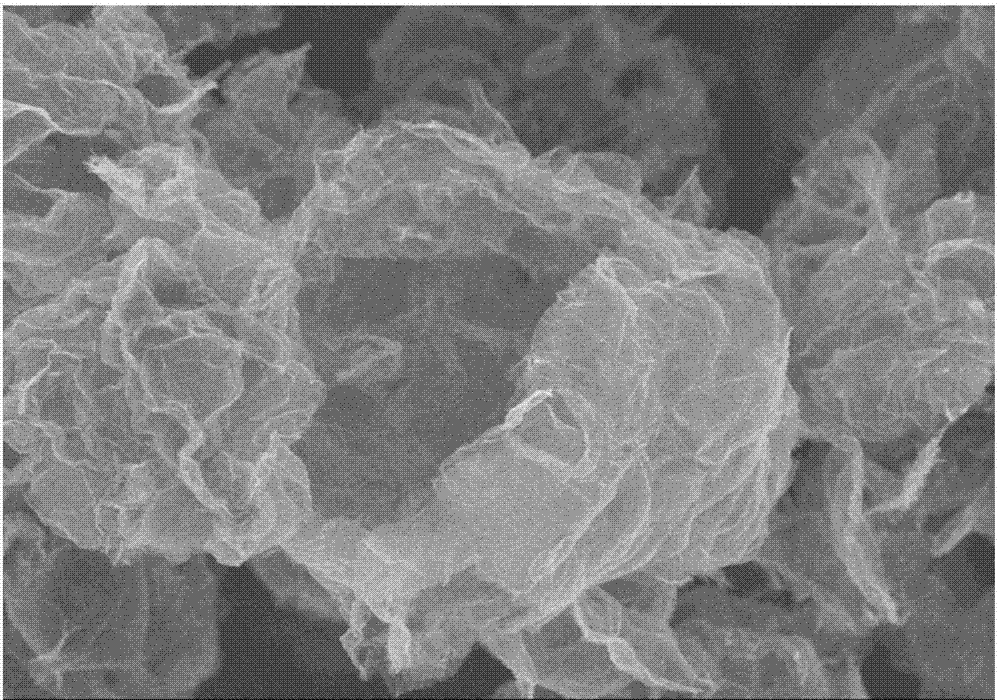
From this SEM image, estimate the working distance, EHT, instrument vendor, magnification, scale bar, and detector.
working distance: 11 mm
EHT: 3 kV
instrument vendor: Hitachi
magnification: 8 K X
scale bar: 5000 nm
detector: SE(M)